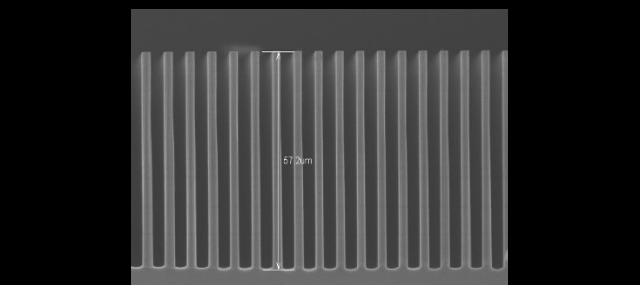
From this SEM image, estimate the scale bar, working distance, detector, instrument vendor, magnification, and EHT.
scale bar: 40000 nm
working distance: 8.2 mm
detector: SE(U)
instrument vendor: Hitachi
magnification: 1.2 K X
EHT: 10 kV